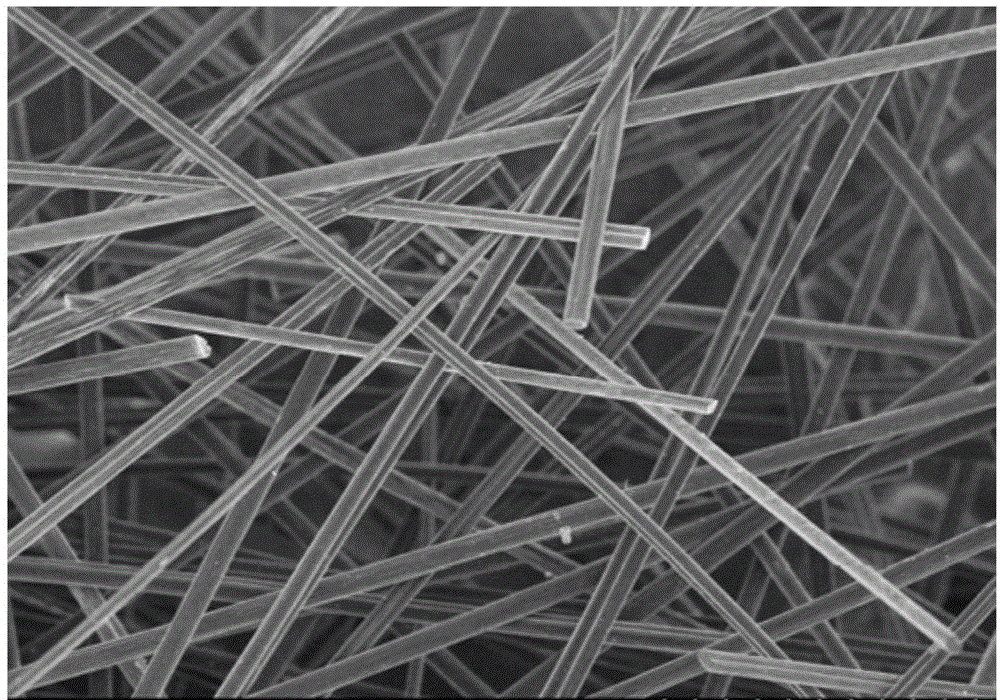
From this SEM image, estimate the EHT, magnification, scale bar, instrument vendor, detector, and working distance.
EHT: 5 kV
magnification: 0.4 K X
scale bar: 100000 nm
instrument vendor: Hitachi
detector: SE(M)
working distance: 8.2 mm